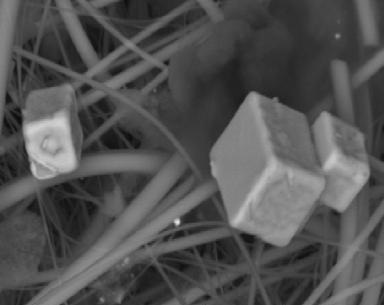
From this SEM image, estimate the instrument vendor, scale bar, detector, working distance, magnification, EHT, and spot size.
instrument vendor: FEI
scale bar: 10000 nm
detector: BSE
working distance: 11.9 mm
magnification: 2 K X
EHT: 20 kV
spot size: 4